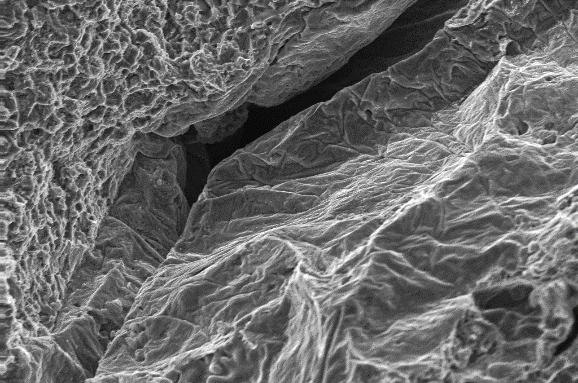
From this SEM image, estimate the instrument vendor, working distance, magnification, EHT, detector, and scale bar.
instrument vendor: Zeiss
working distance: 33.5 mm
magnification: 0.3 K X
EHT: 20 kV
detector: SE1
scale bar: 20000 nm